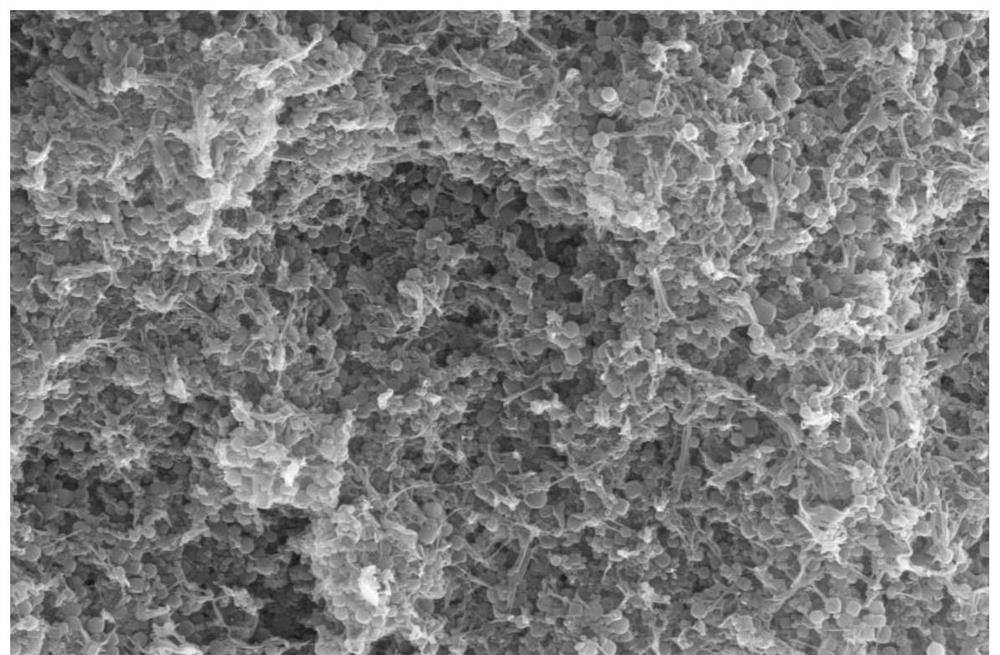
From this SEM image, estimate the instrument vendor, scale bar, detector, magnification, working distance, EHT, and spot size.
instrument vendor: FEI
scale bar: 5000 nm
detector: SE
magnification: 20 K X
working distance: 6.5 mm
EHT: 20 kV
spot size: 3